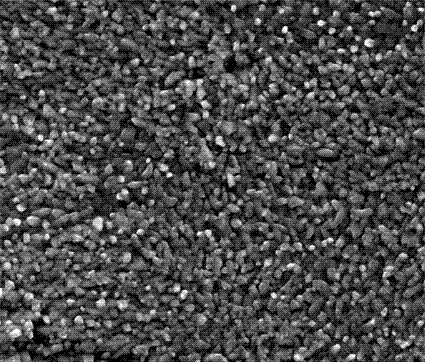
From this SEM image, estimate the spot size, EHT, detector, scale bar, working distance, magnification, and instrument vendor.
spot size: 4.5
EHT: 20 kV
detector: ETD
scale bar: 10000 nm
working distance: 11 mm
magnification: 5 K X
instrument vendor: FEI